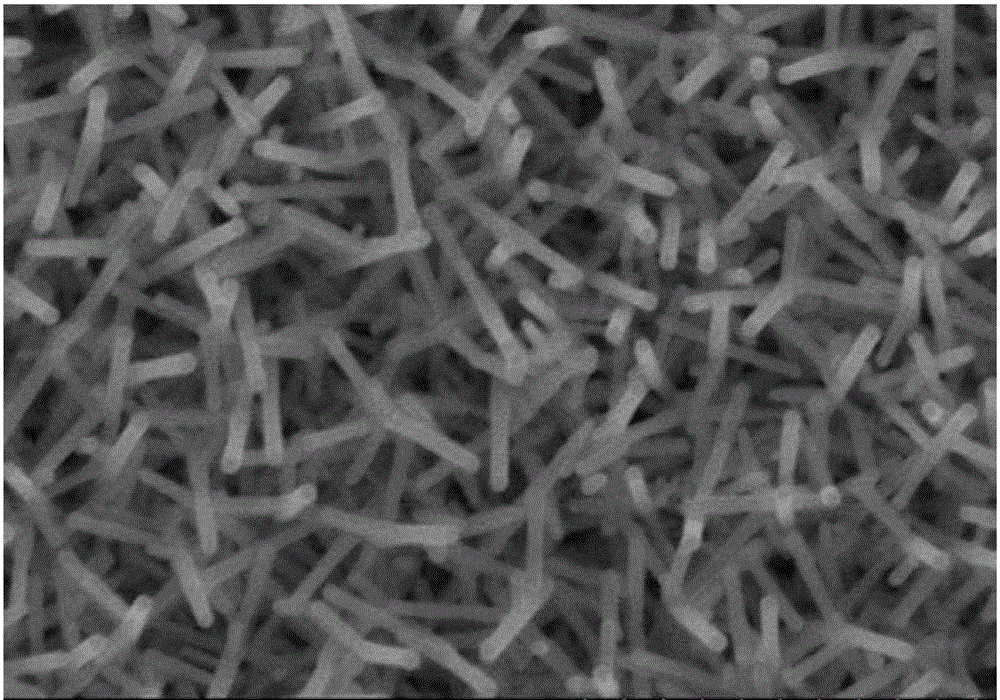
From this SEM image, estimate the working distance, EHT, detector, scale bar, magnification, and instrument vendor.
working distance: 7.6 mm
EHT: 10 kV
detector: SE(M)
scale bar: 1000 nm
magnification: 50 K X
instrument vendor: Hitachi